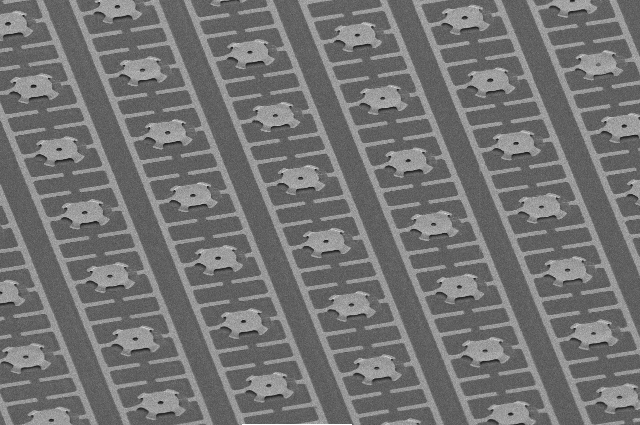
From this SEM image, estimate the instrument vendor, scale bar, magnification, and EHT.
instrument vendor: JEOL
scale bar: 100000 nm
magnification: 0.22 K X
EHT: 5 kV